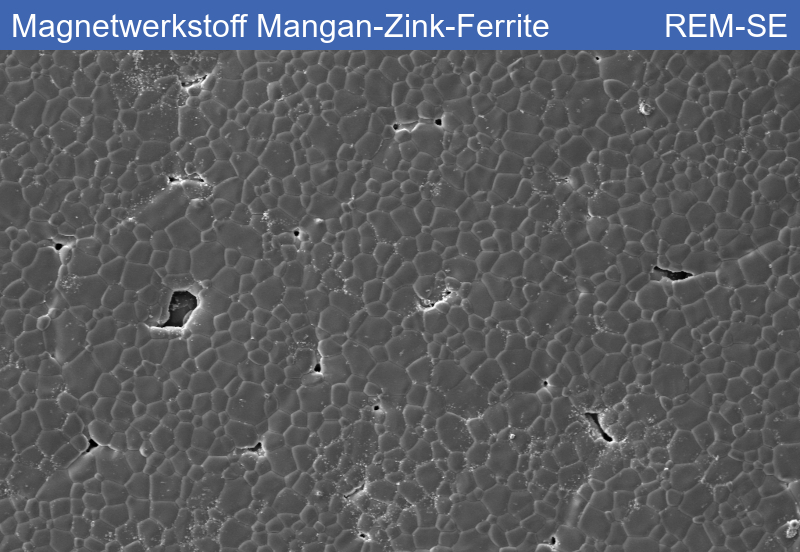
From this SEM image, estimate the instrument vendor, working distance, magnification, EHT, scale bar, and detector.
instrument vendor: Zeiss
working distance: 12.82 mm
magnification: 0.25 K X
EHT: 15 kV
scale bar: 40000 nm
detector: SE1